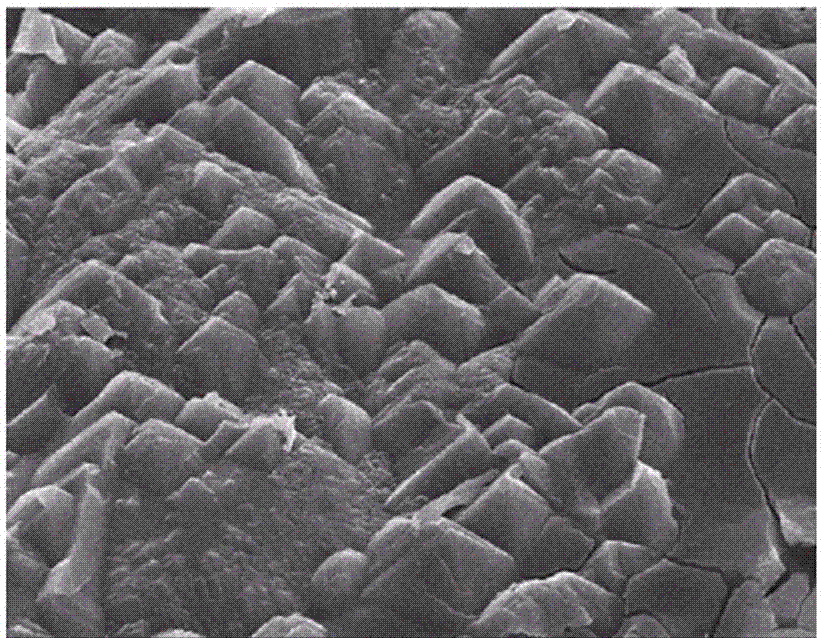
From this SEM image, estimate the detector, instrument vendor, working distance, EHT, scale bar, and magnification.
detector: SEI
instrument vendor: JEOL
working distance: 15.1 mm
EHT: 15 kV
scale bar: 1000 nm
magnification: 3 K X